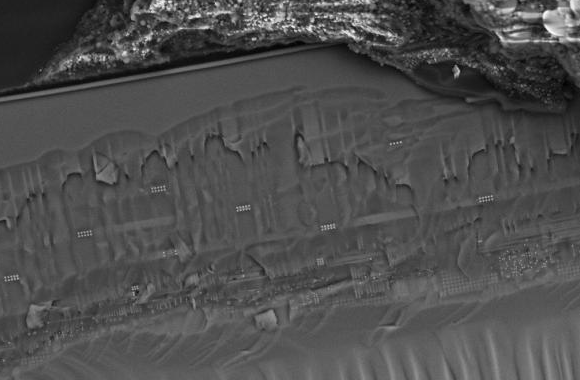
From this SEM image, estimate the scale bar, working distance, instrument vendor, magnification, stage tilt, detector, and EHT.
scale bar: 10000 nm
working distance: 2.5 mm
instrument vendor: Zeiss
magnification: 1.82 K X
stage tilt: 46.8°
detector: InLens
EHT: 15 kV